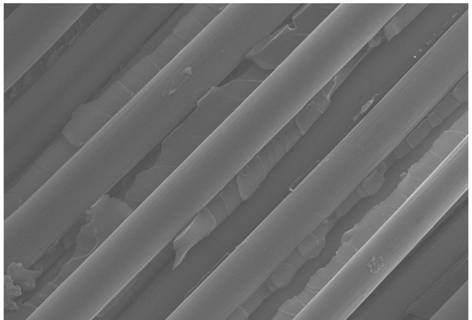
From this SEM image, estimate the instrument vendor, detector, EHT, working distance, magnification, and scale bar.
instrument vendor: Zeiss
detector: InLens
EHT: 20 kV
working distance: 6.4 mm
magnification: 5 K X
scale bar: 10000 nm